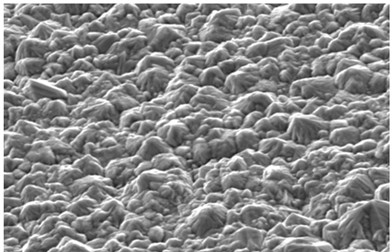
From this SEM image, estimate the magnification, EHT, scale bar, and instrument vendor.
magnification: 2 K X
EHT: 20 kV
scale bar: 10000 nm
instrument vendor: other